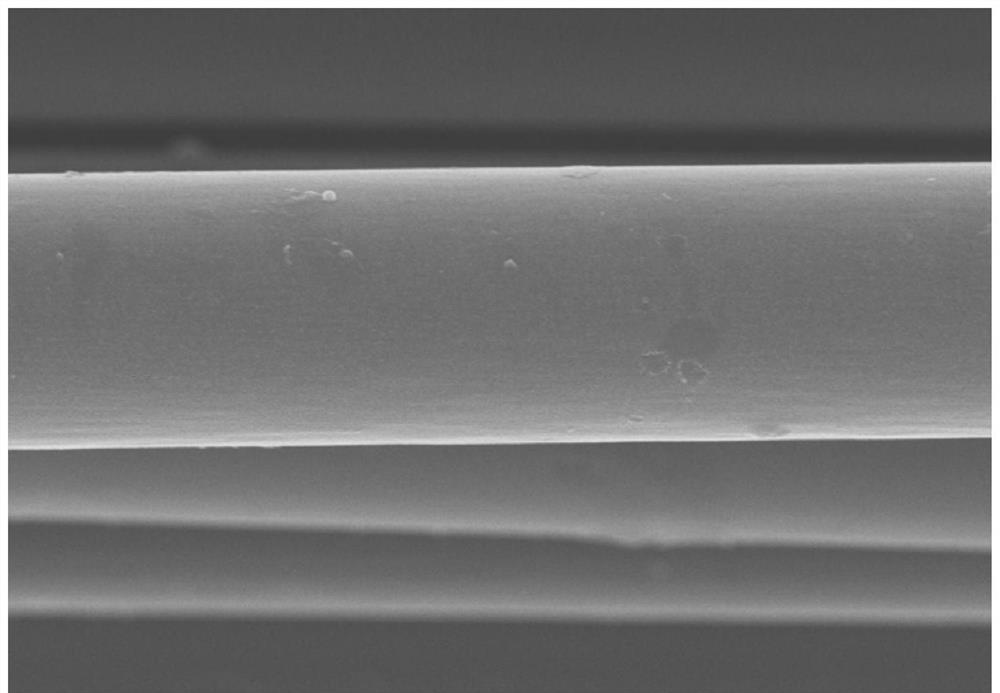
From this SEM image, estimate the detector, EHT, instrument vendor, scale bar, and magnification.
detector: SE(M)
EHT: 8 kV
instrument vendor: Hitachi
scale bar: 10000 nm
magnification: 5 K X